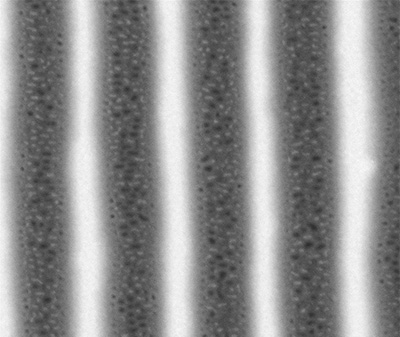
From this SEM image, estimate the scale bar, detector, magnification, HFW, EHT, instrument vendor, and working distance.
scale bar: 500 nm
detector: vCD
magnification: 130 K X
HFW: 2.3 µm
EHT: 1.2 kV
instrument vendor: FEI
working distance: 5.7 mm